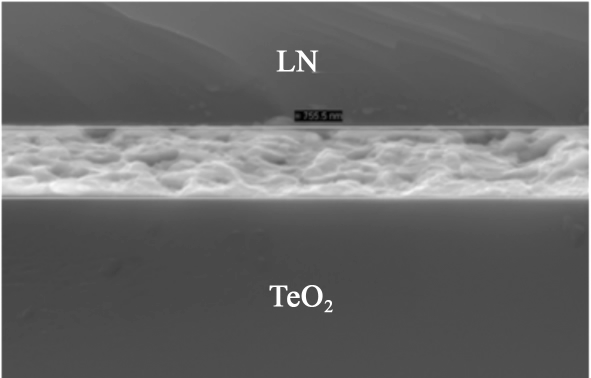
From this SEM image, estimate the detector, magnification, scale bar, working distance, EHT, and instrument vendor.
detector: SE2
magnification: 18.32 K X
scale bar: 300 nm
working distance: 17 mm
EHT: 20 kV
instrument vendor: Zeiss